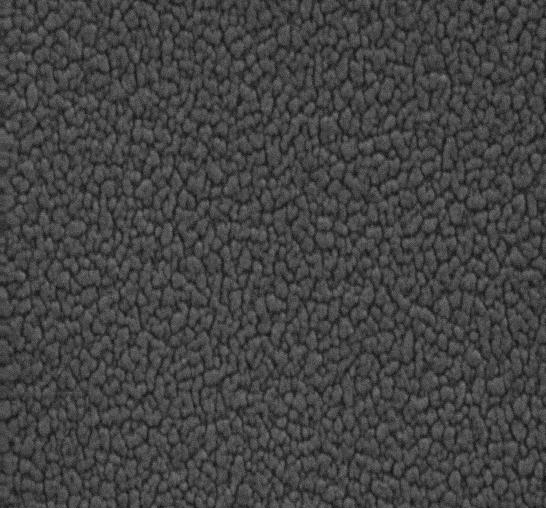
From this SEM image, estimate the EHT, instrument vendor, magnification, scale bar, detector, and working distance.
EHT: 20 kV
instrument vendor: Zeiss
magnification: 30 K X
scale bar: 200 nm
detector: SE1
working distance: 10 mm